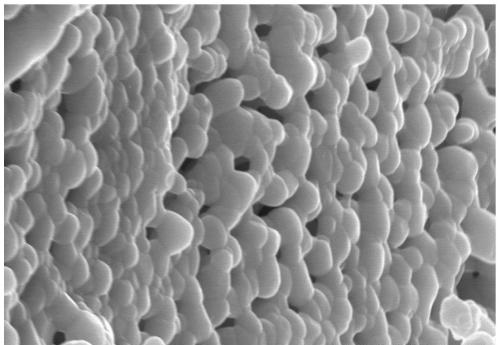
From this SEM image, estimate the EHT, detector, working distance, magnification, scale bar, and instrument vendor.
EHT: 20 kV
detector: SE(U)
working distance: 15.1 mm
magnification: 20 K X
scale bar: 2000 nm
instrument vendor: Hitachi